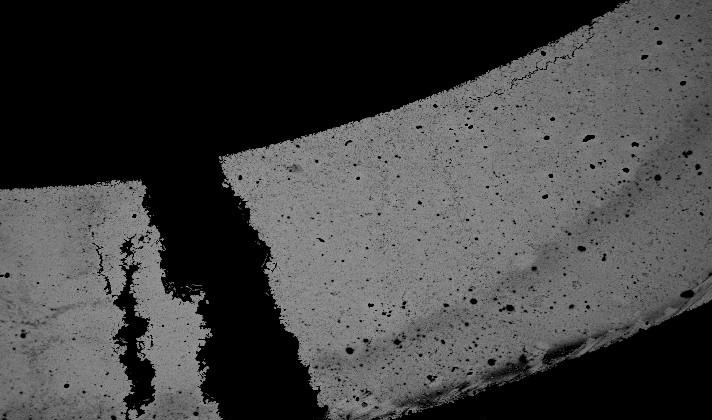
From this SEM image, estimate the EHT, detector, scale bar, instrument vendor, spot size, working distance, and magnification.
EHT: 25 kV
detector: BSE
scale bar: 1000000 nm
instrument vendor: FEI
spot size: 5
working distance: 11.1 mm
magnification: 0.03 K X